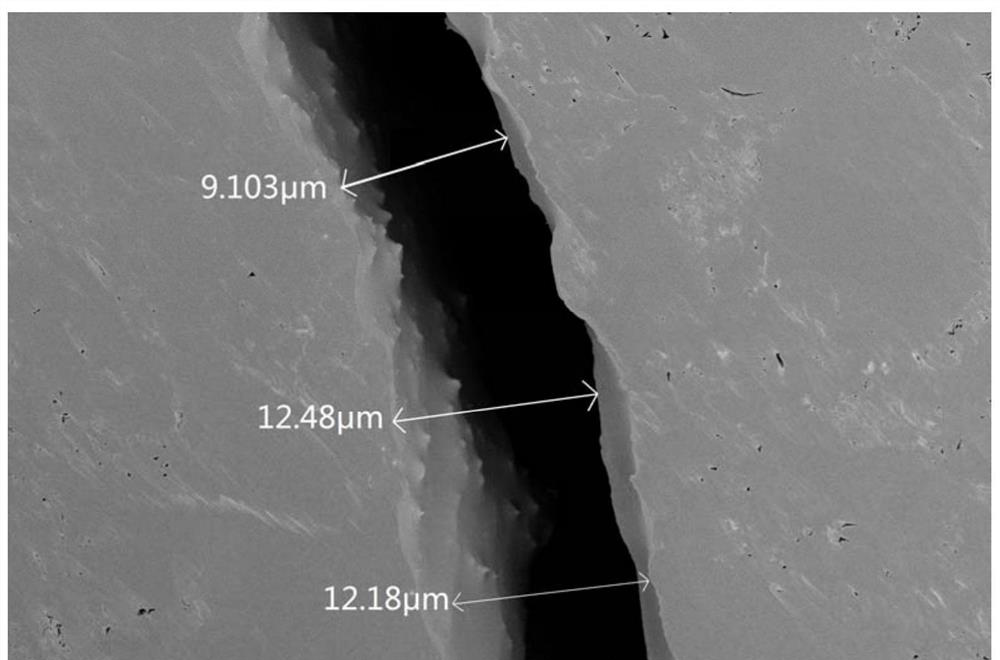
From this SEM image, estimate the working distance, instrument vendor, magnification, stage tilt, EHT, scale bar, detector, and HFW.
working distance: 5 mm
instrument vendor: FEI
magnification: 8.006 K X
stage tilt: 0°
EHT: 2 kV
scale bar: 10000 nm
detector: TLD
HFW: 51.8 µm